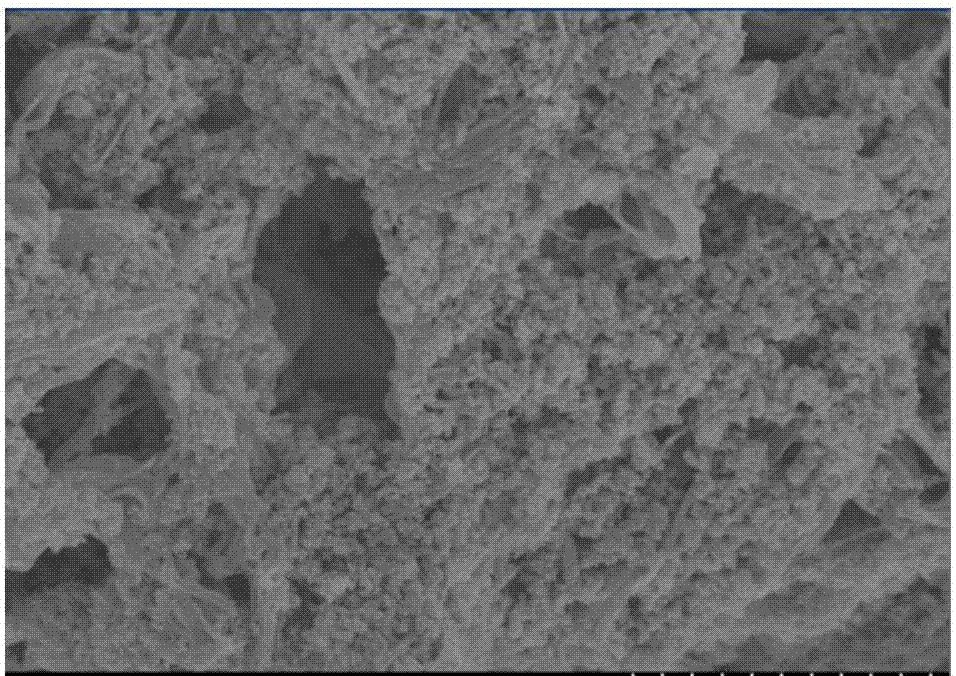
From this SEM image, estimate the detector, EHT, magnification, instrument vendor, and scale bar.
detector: SE(U)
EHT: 4 kV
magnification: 20 K X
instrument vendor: Hitachi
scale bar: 2000 nm